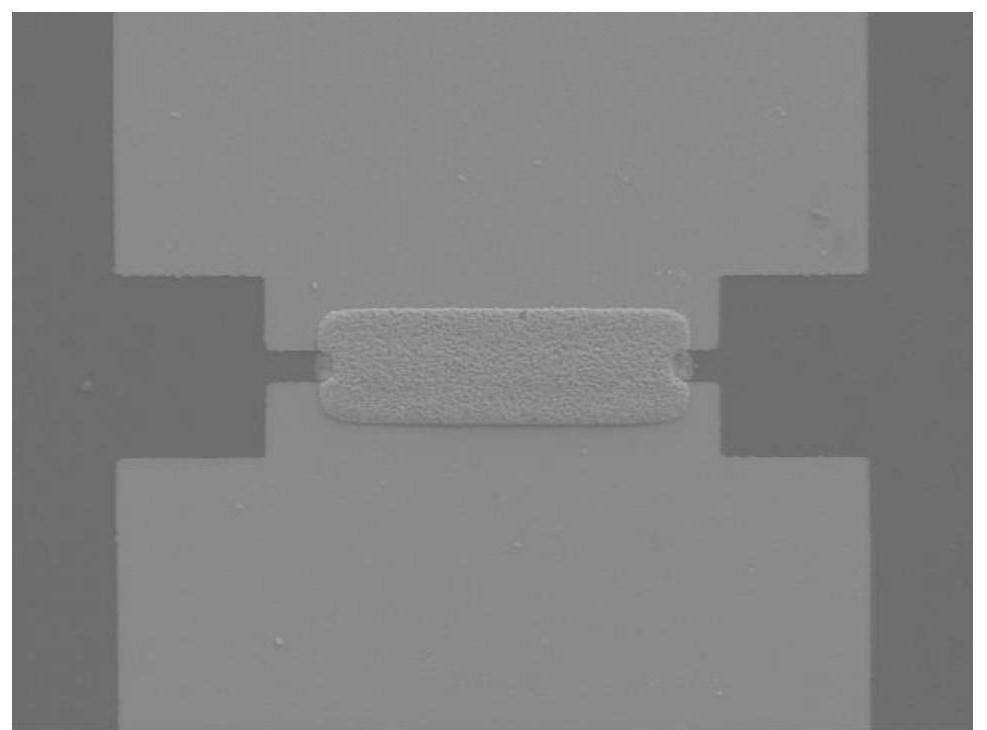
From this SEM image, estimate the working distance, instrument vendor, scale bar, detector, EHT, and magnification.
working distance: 6.4 mm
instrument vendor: JEOL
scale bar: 10000 nm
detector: LED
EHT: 10 kV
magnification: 0.5 K X